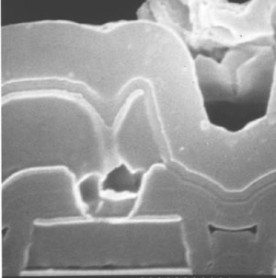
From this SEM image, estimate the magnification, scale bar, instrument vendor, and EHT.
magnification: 24.8 K X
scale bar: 1210 nm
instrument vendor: JEOL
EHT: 20 kV